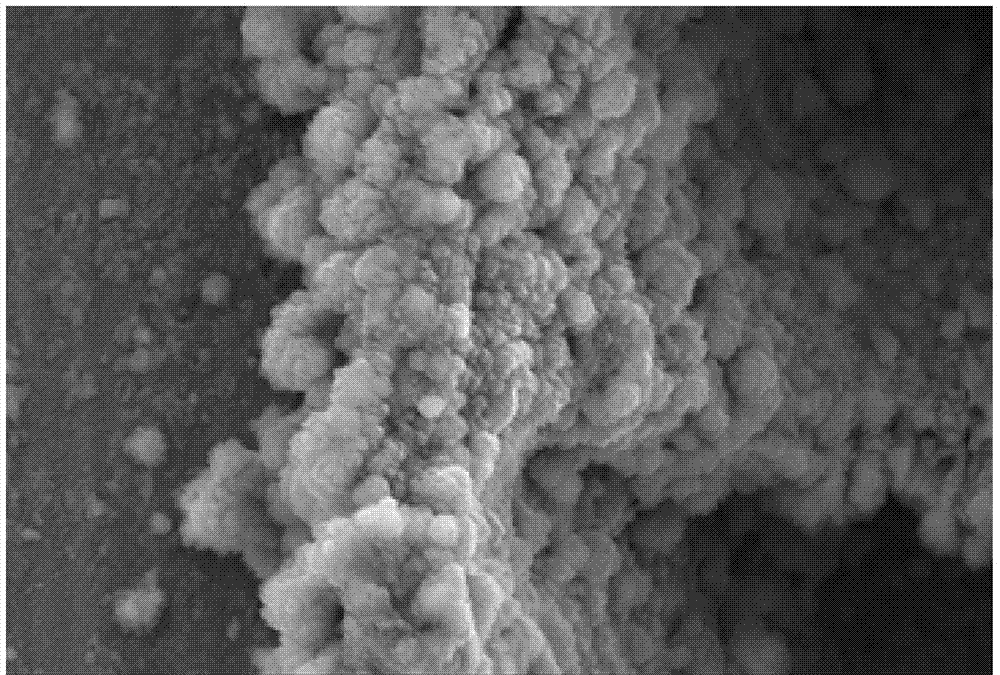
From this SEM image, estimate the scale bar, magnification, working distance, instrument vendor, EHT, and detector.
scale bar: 1000 nm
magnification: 10 K X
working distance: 17.1 mm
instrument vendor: Zeiss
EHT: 15 kV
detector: SE2